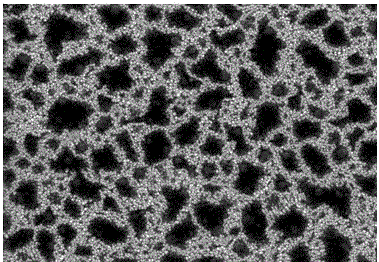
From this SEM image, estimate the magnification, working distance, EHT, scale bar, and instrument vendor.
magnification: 30 K X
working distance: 11.3 mm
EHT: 15 kV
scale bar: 1000 nm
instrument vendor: Hitachi